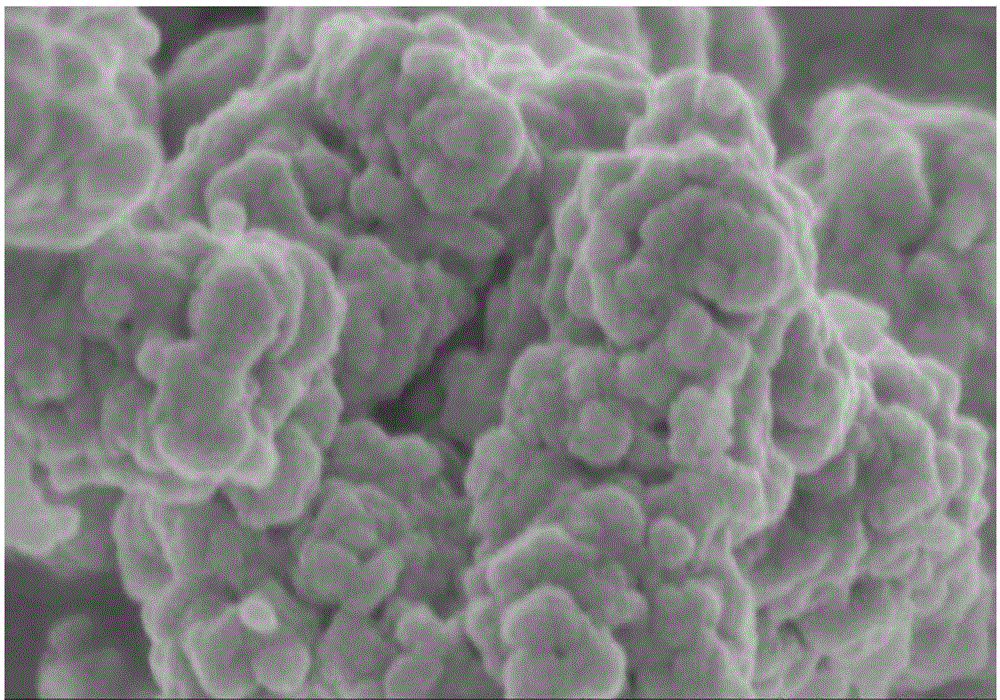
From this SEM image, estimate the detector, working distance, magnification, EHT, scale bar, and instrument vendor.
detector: SE(U)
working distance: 5.9 mm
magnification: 100 K X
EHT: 3 kV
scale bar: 500 nm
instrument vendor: Hitachi